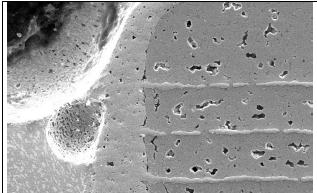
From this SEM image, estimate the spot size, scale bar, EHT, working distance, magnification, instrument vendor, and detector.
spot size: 3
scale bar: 50000 nm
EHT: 20 kV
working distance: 11 mm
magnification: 0.97 K X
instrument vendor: FEI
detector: SE